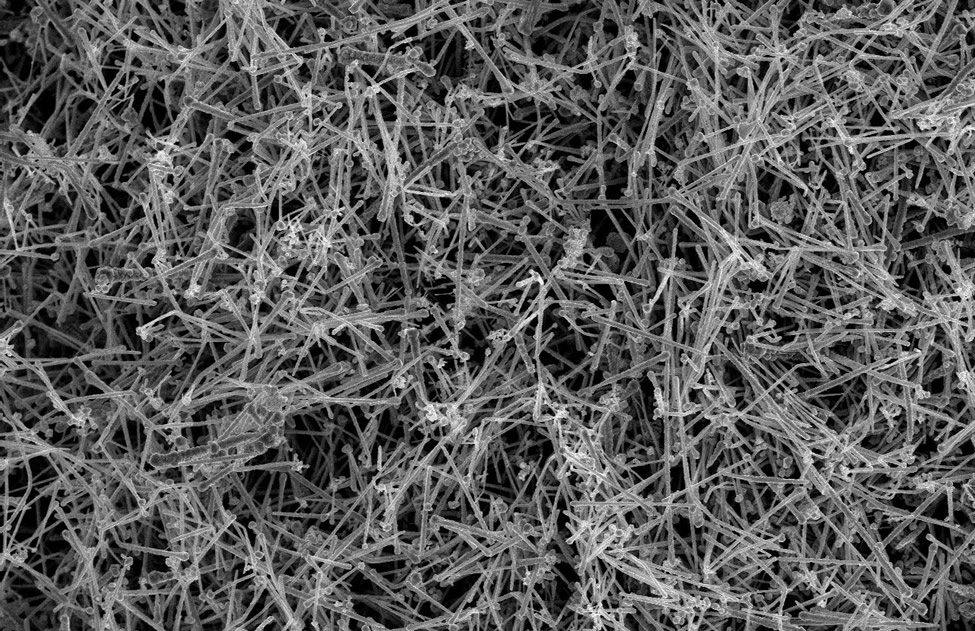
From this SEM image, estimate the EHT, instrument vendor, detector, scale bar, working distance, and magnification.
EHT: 10 kV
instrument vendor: Hitachi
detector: SE(M)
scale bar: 20000 nm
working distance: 10.2 mm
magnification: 2.5 K X